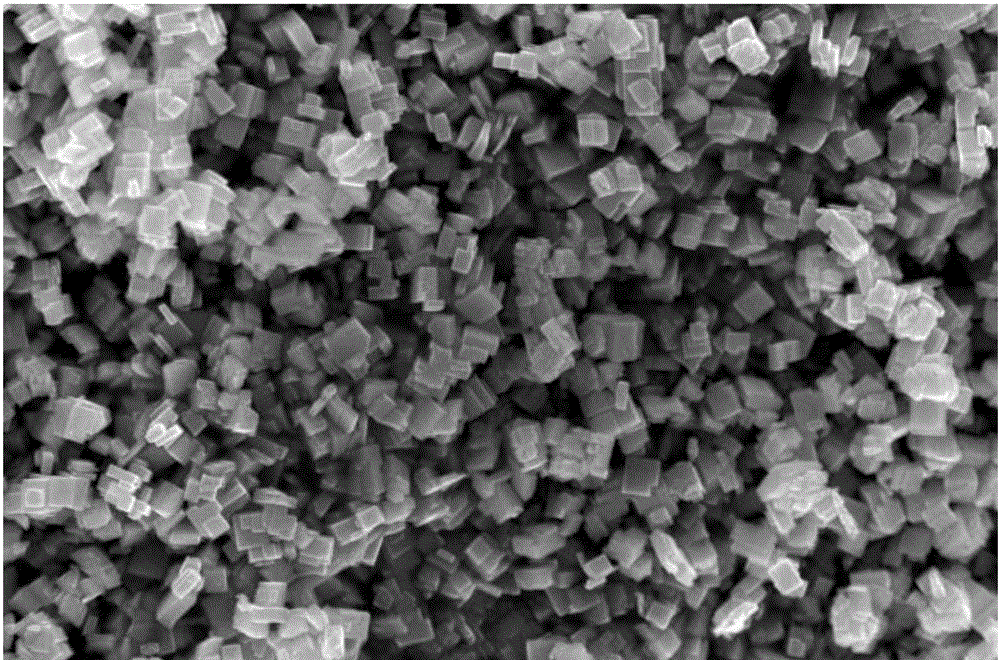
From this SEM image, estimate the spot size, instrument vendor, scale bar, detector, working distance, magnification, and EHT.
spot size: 3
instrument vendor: FEI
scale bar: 1000 nm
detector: TLD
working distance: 5.2 mm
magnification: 40 K X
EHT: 10 kV